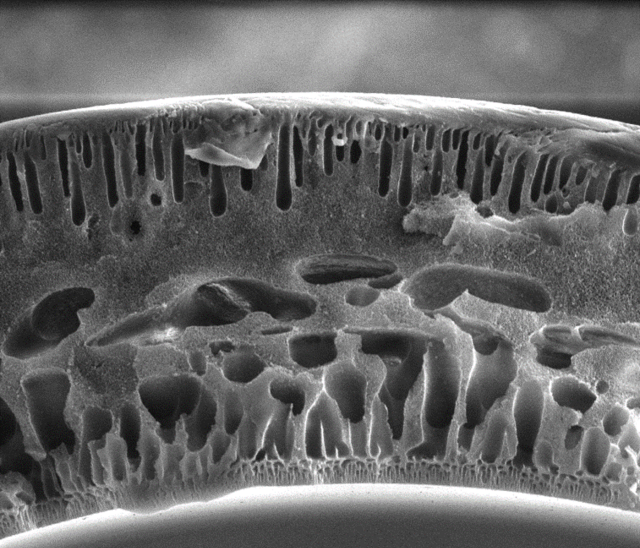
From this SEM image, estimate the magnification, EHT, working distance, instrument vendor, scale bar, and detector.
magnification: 0.647 K X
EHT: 15 kV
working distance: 4 mm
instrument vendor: FEI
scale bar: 100000 nm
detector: ETD